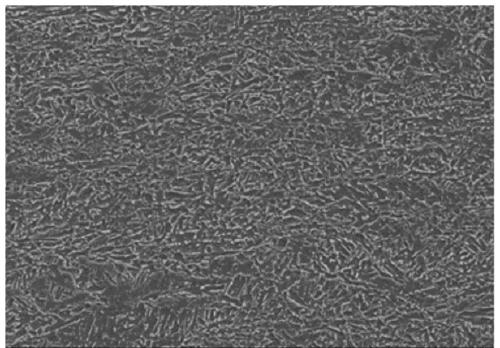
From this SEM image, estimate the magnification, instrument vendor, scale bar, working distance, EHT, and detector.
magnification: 1 K X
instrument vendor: Hitachi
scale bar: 50000 nm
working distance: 6.9 mm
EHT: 15 kV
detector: SE